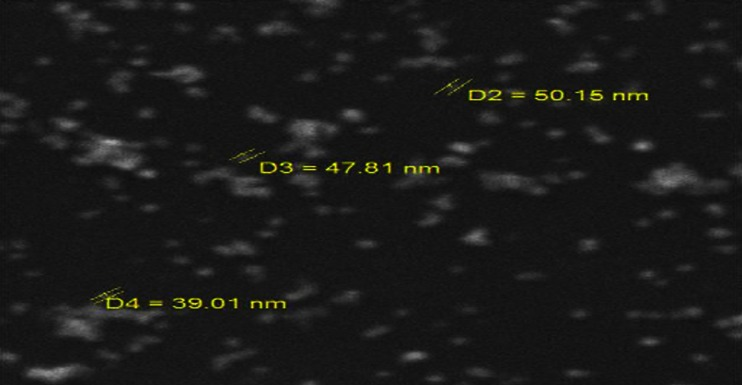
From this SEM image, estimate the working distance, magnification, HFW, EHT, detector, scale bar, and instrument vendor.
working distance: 8.52 mm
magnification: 41.3 K X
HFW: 3.08 µm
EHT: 20 kV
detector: SE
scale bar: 500 nm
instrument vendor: Tescan